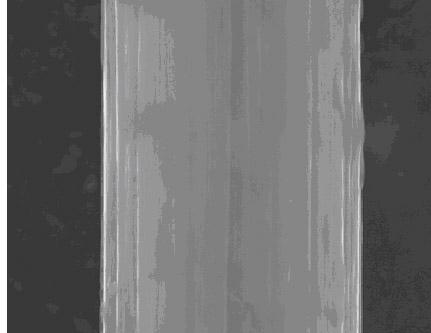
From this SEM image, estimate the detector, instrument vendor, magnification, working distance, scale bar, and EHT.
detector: SE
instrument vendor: FEI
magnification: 35 K X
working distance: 12 mm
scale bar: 3000 nm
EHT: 20 kV